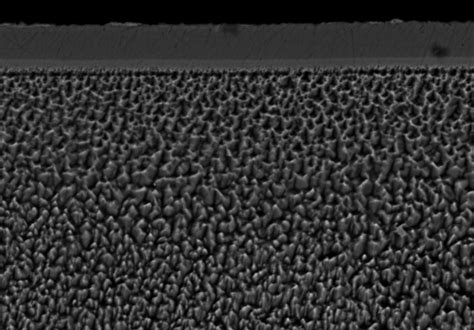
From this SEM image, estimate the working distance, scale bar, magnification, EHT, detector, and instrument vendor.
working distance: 10 mm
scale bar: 10000 nm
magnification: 3 K X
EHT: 15 kV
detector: YAGBSE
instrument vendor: Hitachi